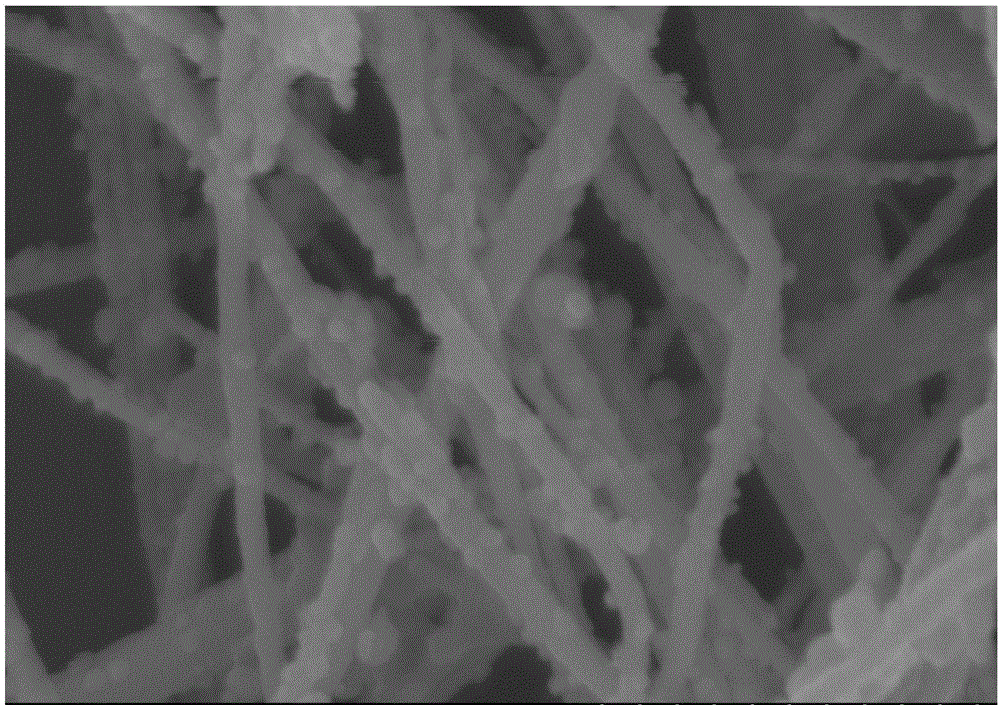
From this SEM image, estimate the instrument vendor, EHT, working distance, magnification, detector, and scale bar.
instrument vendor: Hitachi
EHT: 5 kV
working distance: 8 mm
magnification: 120 K X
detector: SE(M)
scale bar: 400 nm